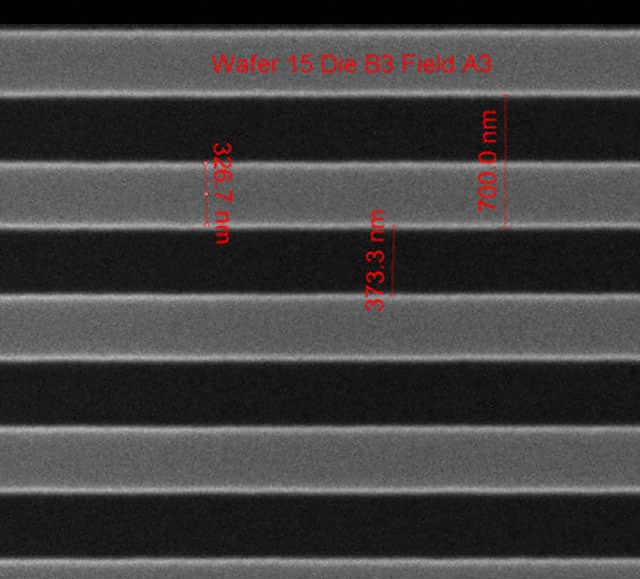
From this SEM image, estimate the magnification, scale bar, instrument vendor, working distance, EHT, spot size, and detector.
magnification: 75.019 K X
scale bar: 1000 nm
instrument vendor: FEI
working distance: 10.2 mm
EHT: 10 kV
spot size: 1.5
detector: ETD